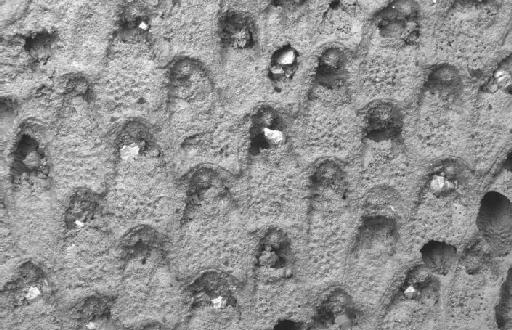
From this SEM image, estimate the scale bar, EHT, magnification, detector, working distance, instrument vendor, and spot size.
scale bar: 100000 nm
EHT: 20 kV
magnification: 0.15 K X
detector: QBSD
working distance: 15 mm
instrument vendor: Zeiss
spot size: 500